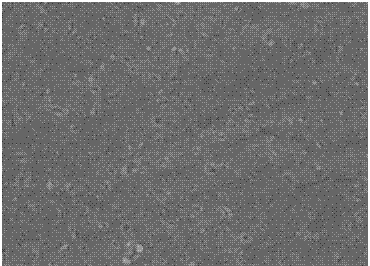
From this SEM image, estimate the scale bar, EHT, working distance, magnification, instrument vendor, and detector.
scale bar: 20000 nm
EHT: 20 kV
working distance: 15.59 mm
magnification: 3 K X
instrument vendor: Tescan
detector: SE